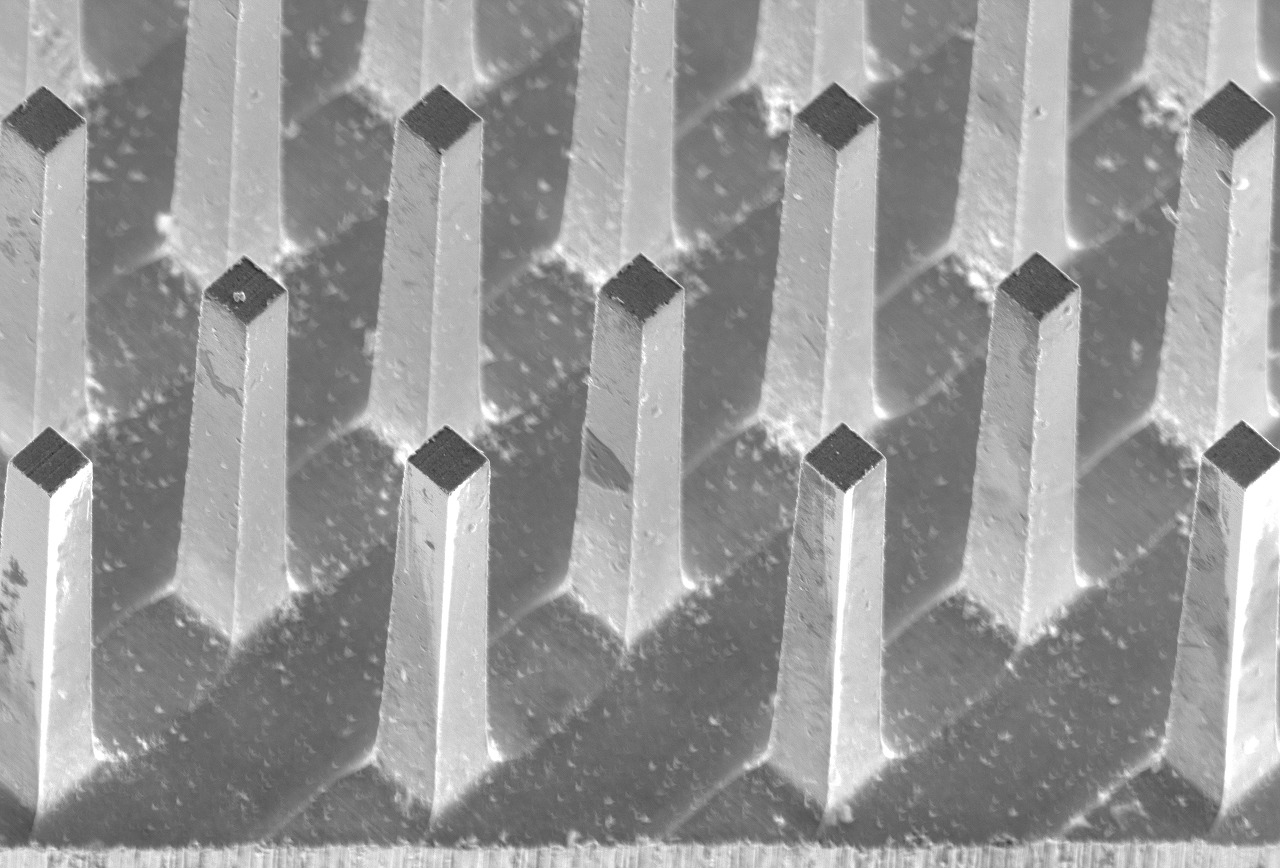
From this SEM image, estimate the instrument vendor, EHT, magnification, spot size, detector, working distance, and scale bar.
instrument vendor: JEOL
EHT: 20 kV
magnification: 0.3 K X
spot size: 60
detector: SEI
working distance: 13 mm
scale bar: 50000 nm